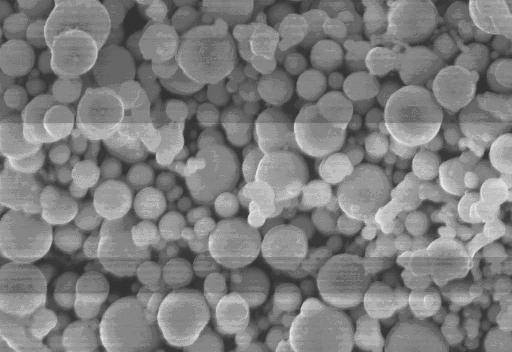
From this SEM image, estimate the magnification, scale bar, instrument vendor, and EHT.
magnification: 5 K X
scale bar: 10000 nm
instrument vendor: other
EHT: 20 kV